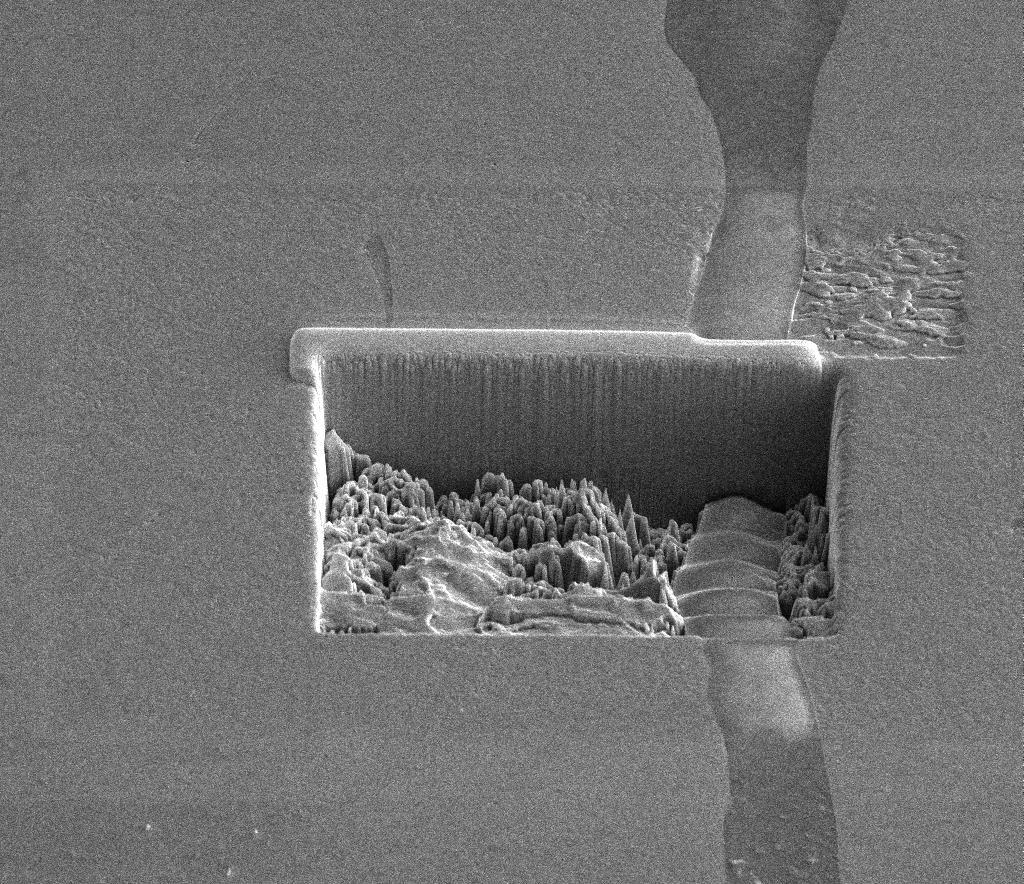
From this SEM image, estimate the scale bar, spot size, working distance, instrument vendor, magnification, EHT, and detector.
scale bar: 20000 nm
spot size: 4.5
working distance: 10 mm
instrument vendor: FEI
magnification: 2.5 K X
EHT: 10 kV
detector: ETD